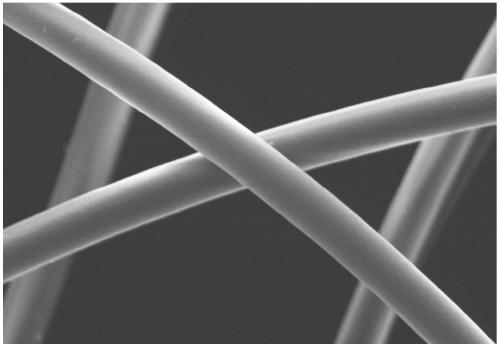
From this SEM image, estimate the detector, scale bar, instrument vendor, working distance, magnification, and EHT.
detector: SE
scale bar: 10000 nm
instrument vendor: Hitachi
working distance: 11.1 mm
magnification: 3 K X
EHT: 15 kV